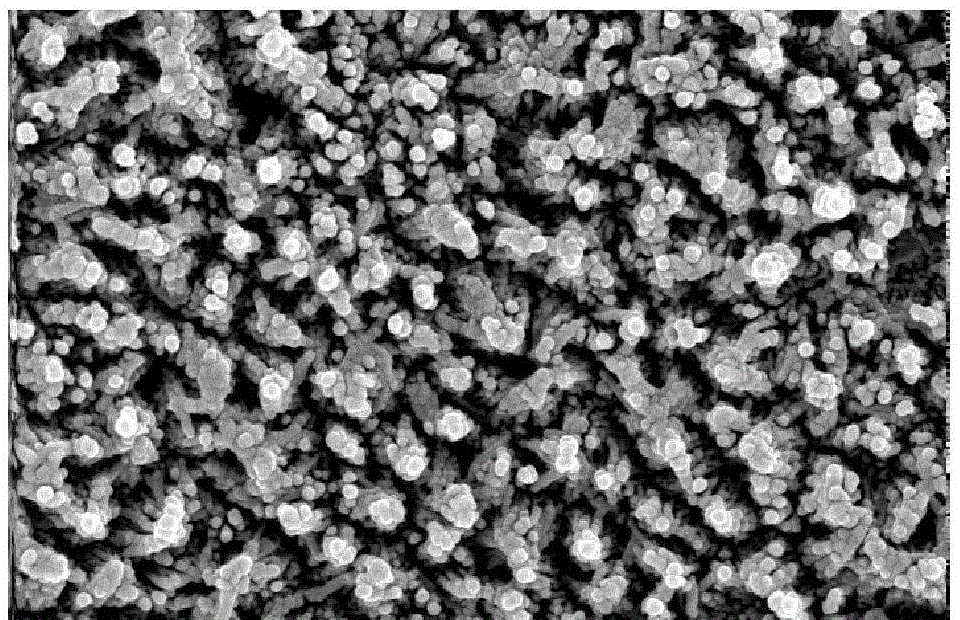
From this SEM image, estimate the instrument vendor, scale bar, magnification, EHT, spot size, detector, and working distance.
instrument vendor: FEI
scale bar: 1000 nm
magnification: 50 K X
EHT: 20 kV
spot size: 3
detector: TLD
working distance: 6.4 mm